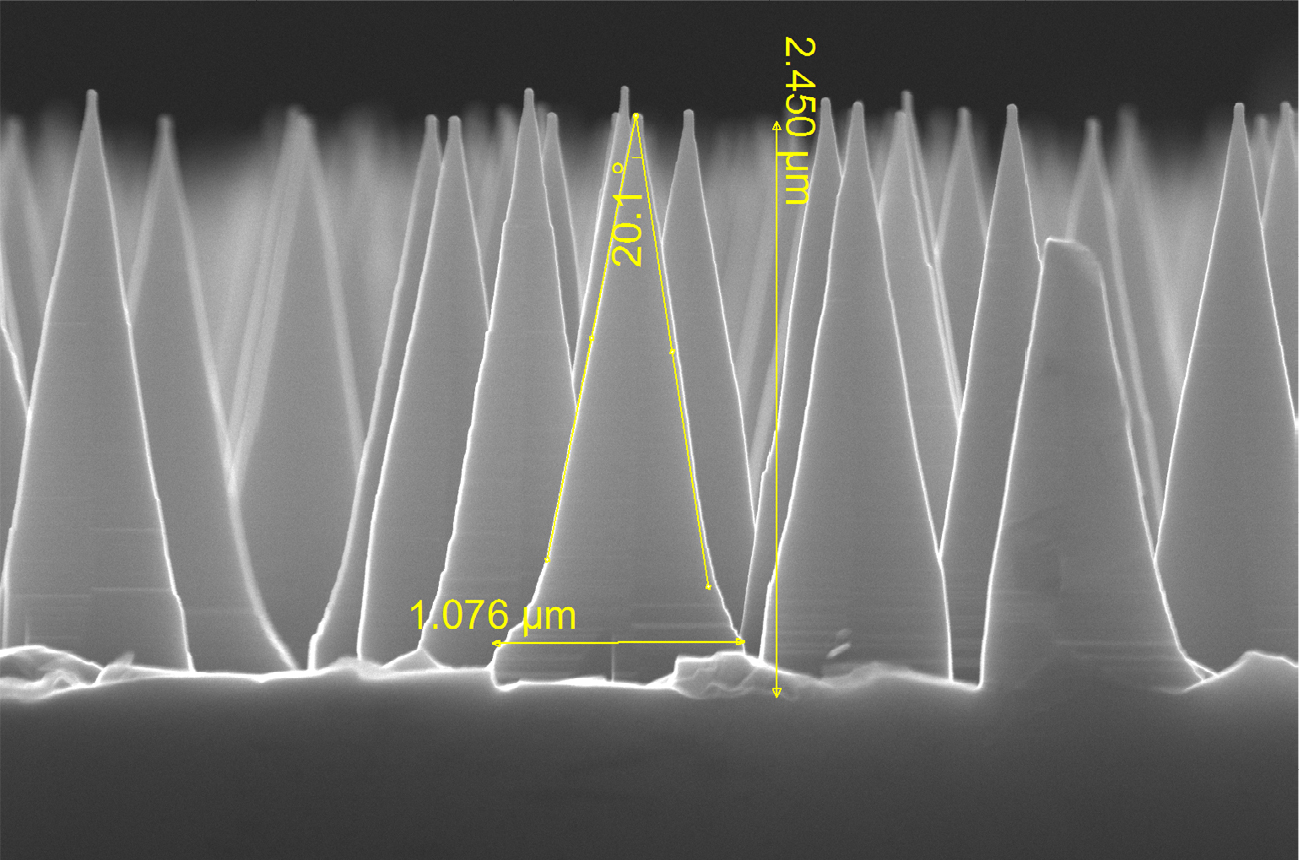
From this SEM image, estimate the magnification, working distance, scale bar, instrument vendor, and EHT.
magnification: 75 K X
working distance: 5.9 mm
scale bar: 2000 nm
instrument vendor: FEI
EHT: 30 kV